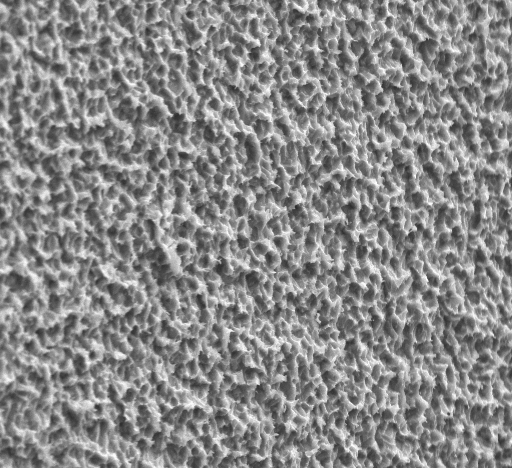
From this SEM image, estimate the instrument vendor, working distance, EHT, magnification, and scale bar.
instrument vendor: JEOL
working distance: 11 mm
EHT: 20 kV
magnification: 2 K X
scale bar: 20000 nm